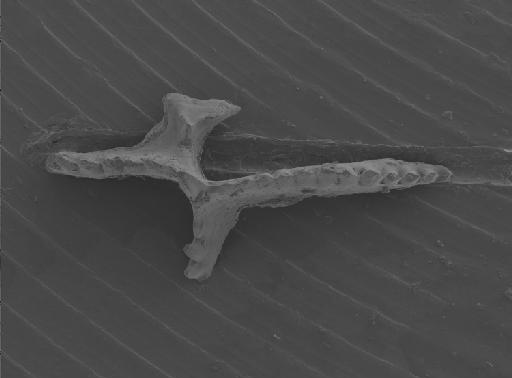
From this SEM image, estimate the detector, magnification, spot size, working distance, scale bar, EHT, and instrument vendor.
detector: SE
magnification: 0.1 K X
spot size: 3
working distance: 10.2 mm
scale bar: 200000 nm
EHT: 5 kV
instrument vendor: FEI